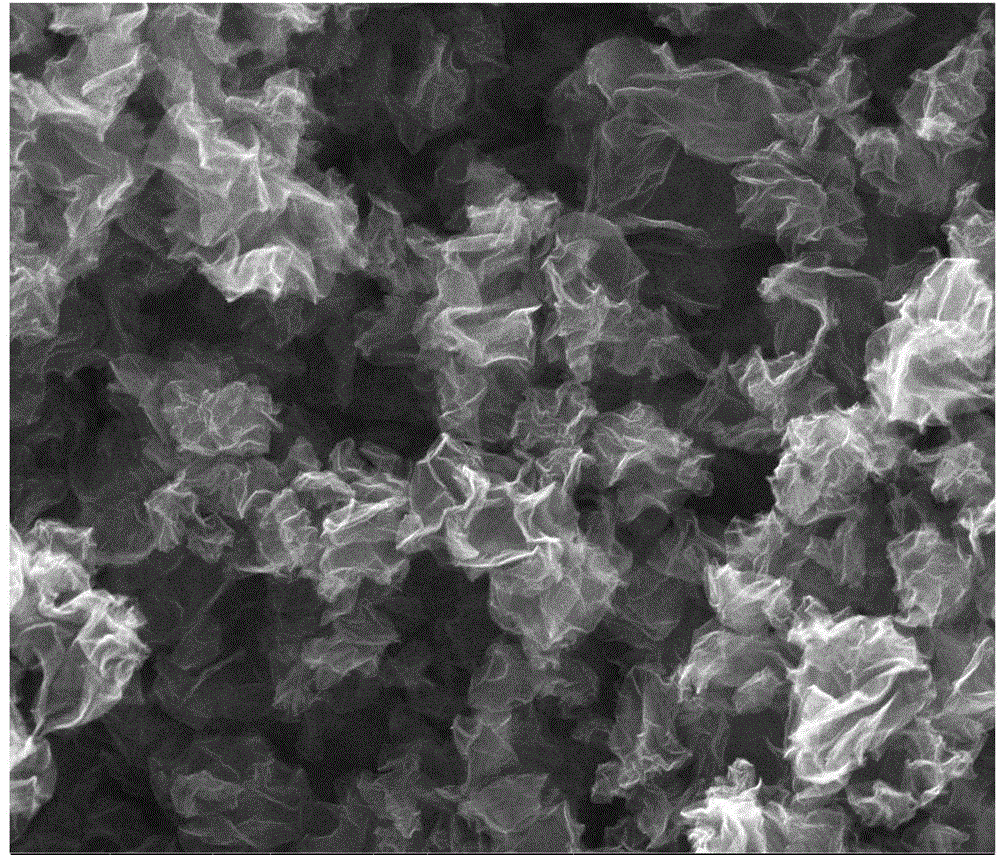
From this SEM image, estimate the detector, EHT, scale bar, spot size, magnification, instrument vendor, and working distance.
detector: ETD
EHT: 20 kV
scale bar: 2000 nm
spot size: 3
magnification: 60 K X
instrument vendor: FEI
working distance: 10 mm